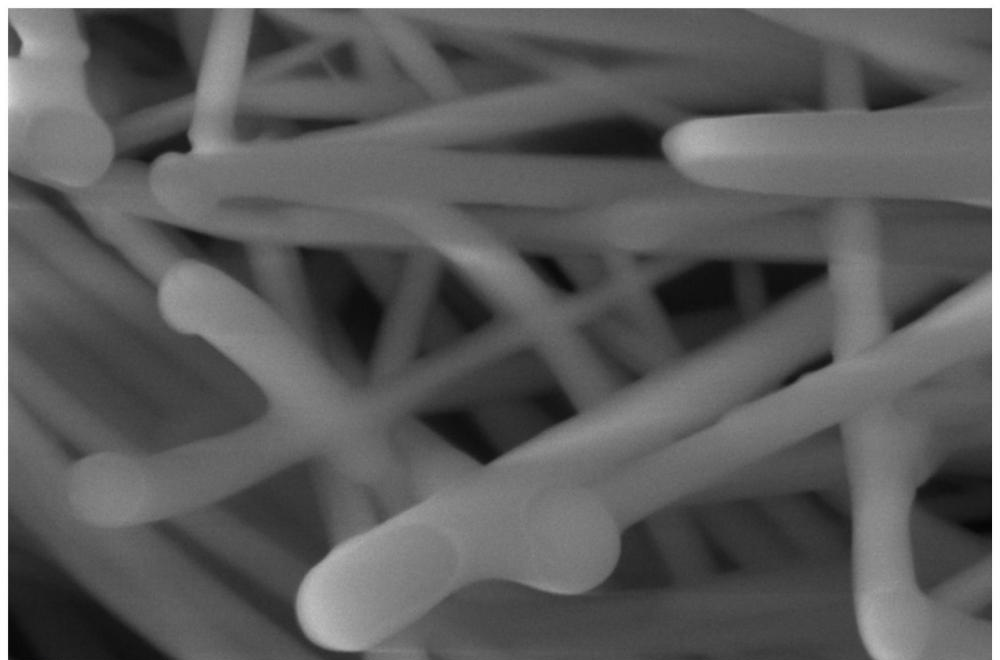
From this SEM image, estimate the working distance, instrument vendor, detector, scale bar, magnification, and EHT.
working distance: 11.4 mm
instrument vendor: Thermo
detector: ETD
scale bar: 1000 nm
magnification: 100 K X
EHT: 20 kV